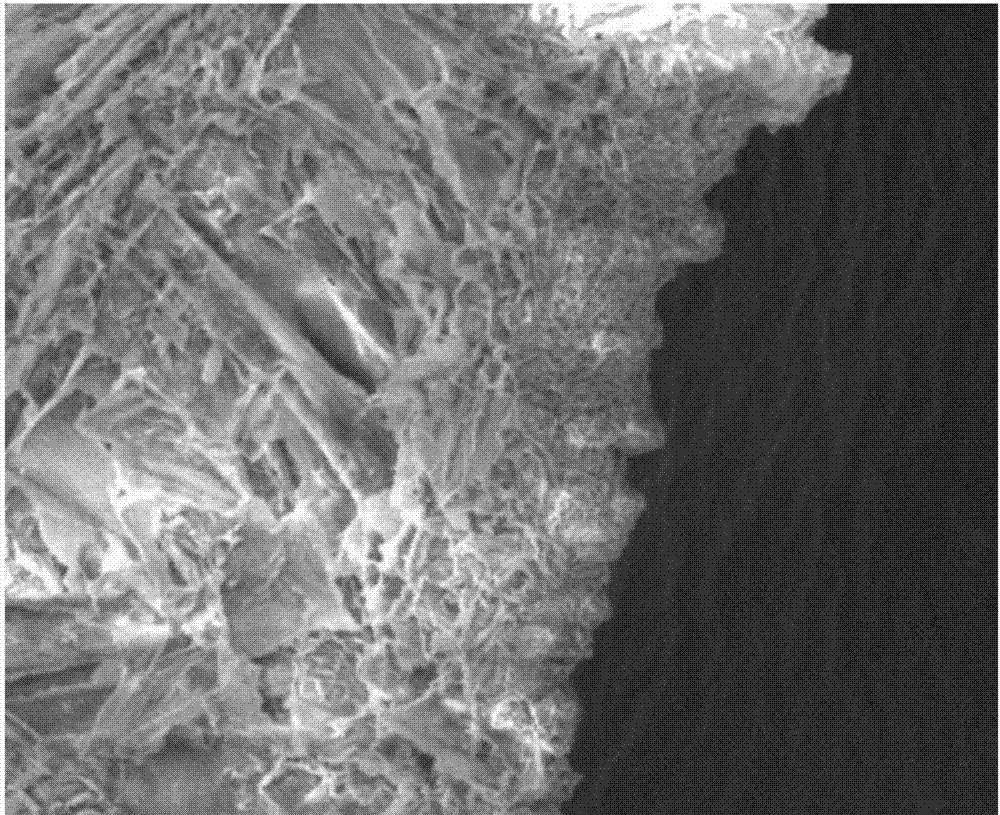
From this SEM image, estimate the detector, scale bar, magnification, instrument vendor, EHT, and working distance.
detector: SEI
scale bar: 10000 nm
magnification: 1 K X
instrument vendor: JEOL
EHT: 5 kV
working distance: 7 mm